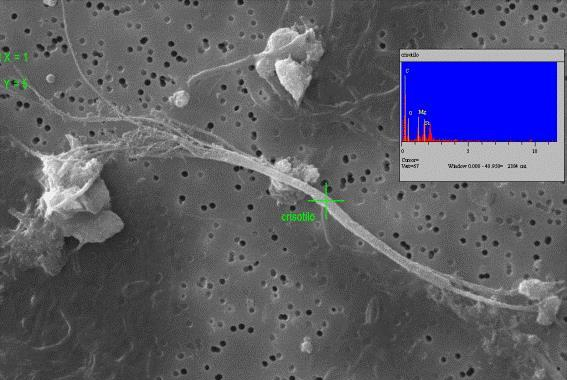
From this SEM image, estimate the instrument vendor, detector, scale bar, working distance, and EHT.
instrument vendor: Zeiss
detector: SE1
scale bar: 10000 nm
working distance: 15 mm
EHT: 20 kV